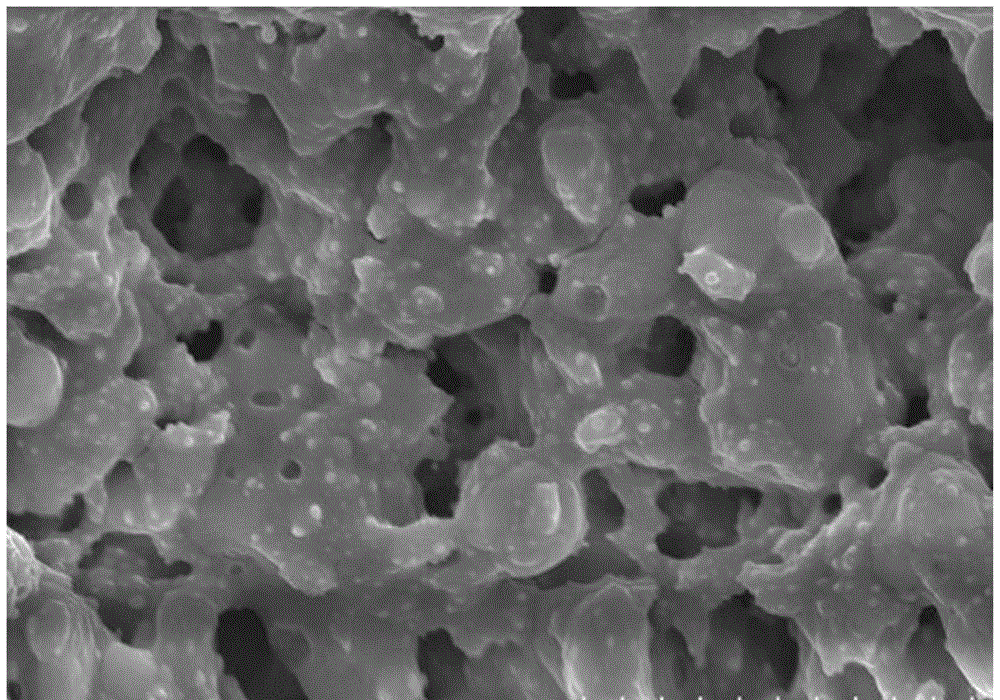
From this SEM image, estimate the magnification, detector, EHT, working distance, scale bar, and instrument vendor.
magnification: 10 K X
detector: SE(M)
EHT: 15 kV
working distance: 8.3 mm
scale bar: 5000 nm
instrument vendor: Hitachi